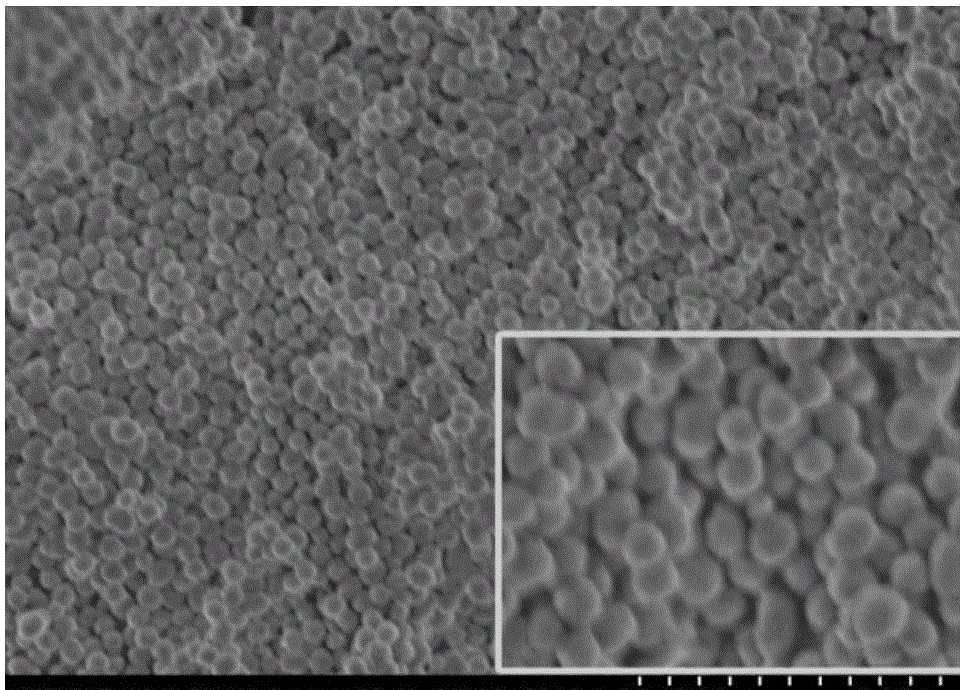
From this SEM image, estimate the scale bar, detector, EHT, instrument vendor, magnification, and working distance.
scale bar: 2000 nm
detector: SE(M)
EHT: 2 kV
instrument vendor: Hitachi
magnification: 20 K X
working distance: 5.3 mm